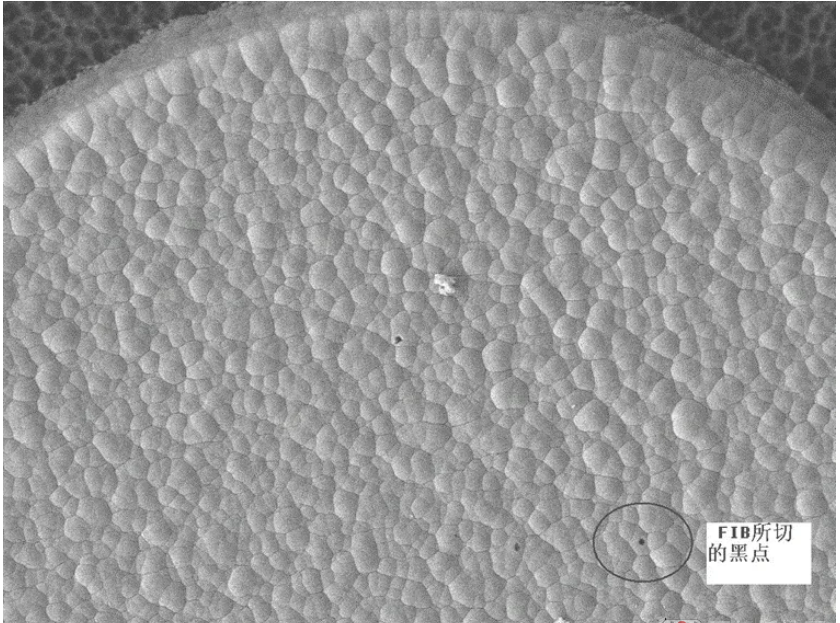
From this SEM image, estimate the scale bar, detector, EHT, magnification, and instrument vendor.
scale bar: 10000 nm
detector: LEI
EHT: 10 kV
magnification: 0.8 K X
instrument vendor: JEOL